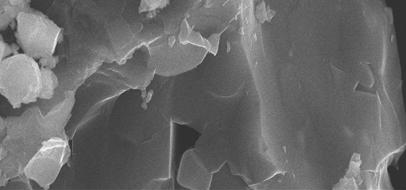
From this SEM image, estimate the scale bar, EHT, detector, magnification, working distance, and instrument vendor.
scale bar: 1000 nm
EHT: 20 kV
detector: SEI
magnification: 5 K X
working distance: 15 mm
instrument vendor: JEOL